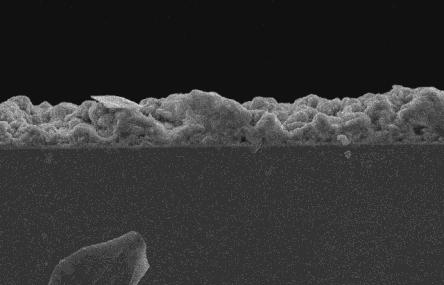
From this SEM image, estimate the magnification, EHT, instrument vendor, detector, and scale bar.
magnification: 1 K X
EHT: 20 kV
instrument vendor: JEOL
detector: SEI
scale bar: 10000 nm